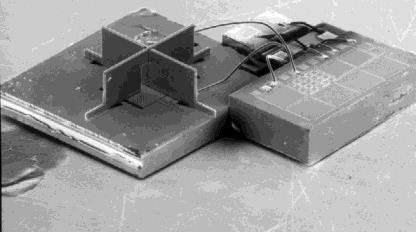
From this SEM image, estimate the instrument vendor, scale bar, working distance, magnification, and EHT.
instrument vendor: JEOL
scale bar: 1000000 nm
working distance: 29 mm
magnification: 0.019 K X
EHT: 30 kV